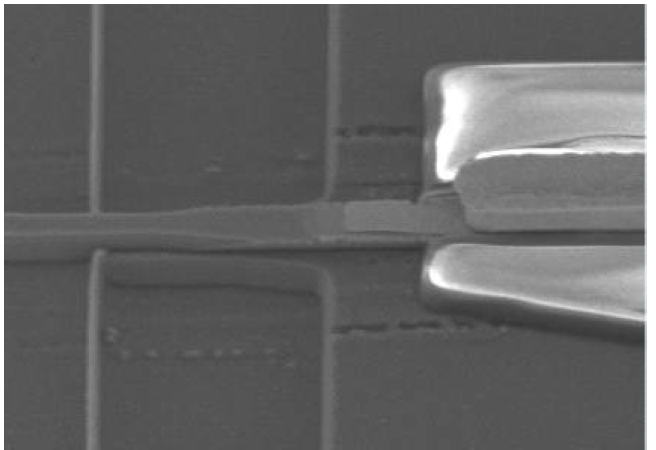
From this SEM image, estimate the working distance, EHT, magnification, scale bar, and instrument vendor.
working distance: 10.9 mm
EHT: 10 kV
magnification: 2 K X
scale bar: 20000 nm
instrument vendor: Hitachi